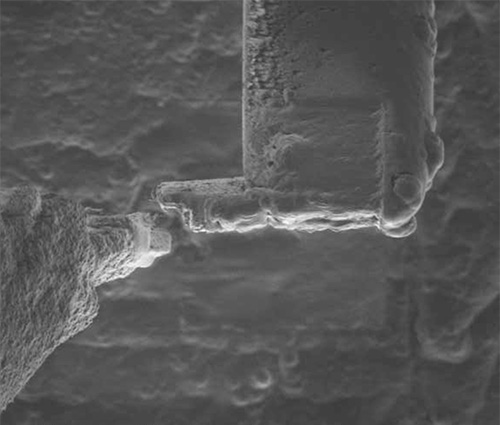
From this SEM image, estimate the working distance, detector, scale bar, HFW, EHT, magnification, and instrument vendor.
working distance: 18 mm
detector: SED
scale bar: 20000 nm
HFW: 122 µm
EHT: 30 kV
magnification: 2.5 K X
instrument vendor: FEI